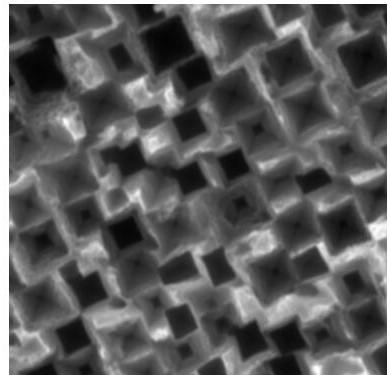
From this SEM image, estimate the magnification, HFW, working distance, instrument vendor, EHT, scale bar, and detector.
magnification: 20 K X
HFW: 10.4 µm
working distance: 15.13 mm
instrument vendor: Tescan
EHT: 20 kV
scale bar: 2000 nm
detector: SE